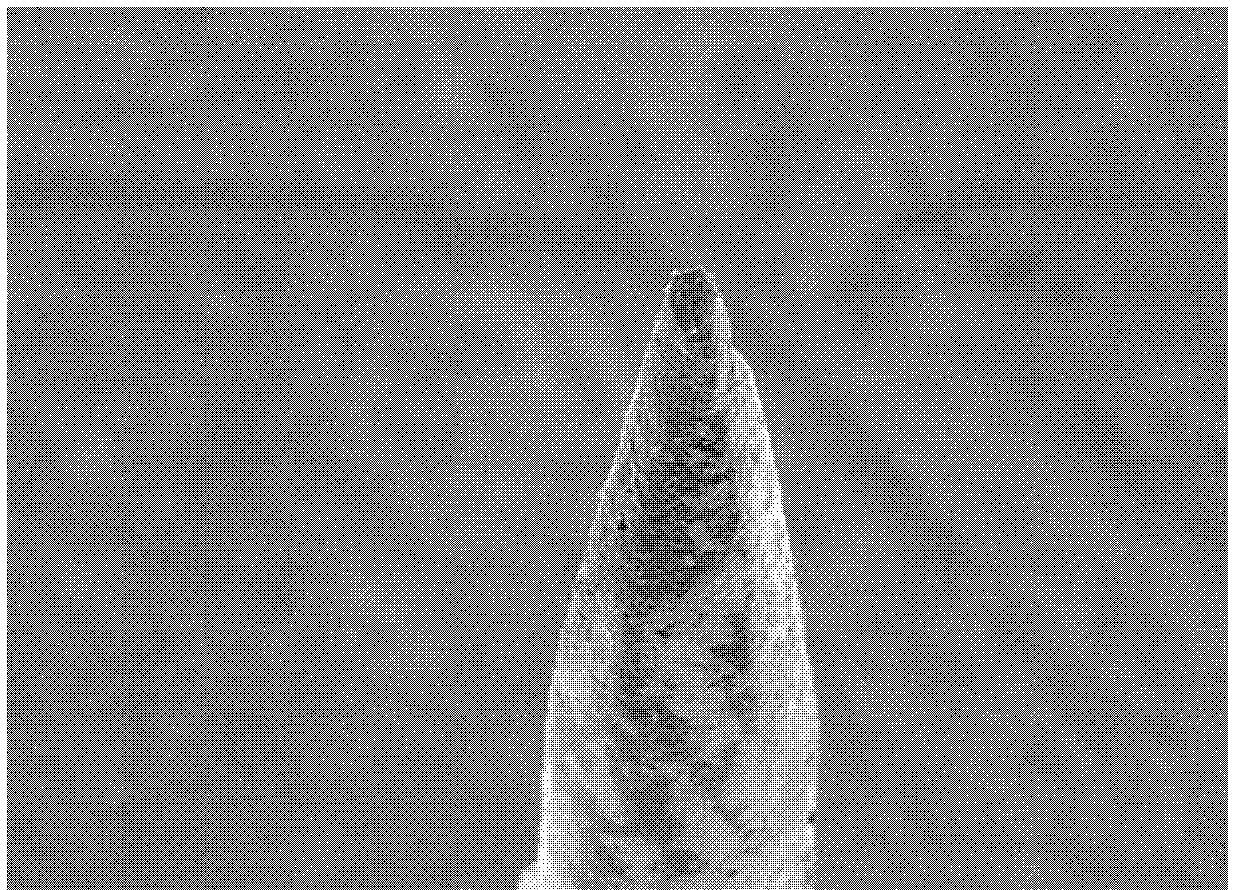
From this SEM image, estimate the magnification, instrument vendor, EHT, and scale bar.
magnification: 0.07 K X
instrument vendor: JEOL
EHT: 10 kV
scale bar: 200000 nm